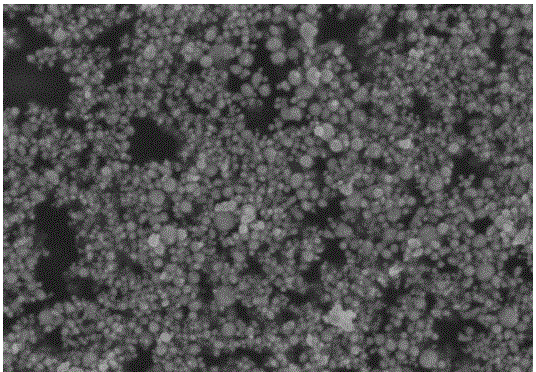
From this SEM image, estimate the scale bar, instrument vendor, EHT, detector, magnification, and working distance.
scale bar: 1000 nm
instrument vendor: Hitachi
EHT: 3 kV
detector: SE(U,LA0)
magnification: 50 K X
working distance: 8.9 mm